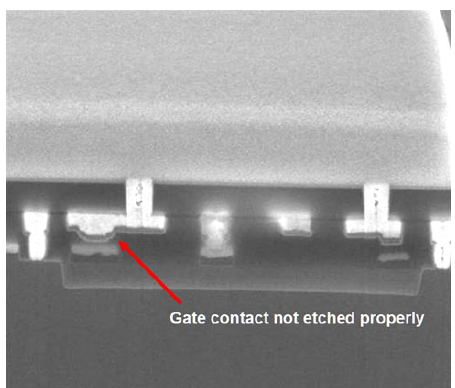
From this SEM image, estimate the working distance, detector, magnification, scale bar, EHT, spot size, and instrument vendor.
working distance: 4.721 mm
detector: TLD-D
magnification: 80 K X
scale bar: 1000 nm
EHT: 10 kV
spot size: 3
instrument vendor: FEI